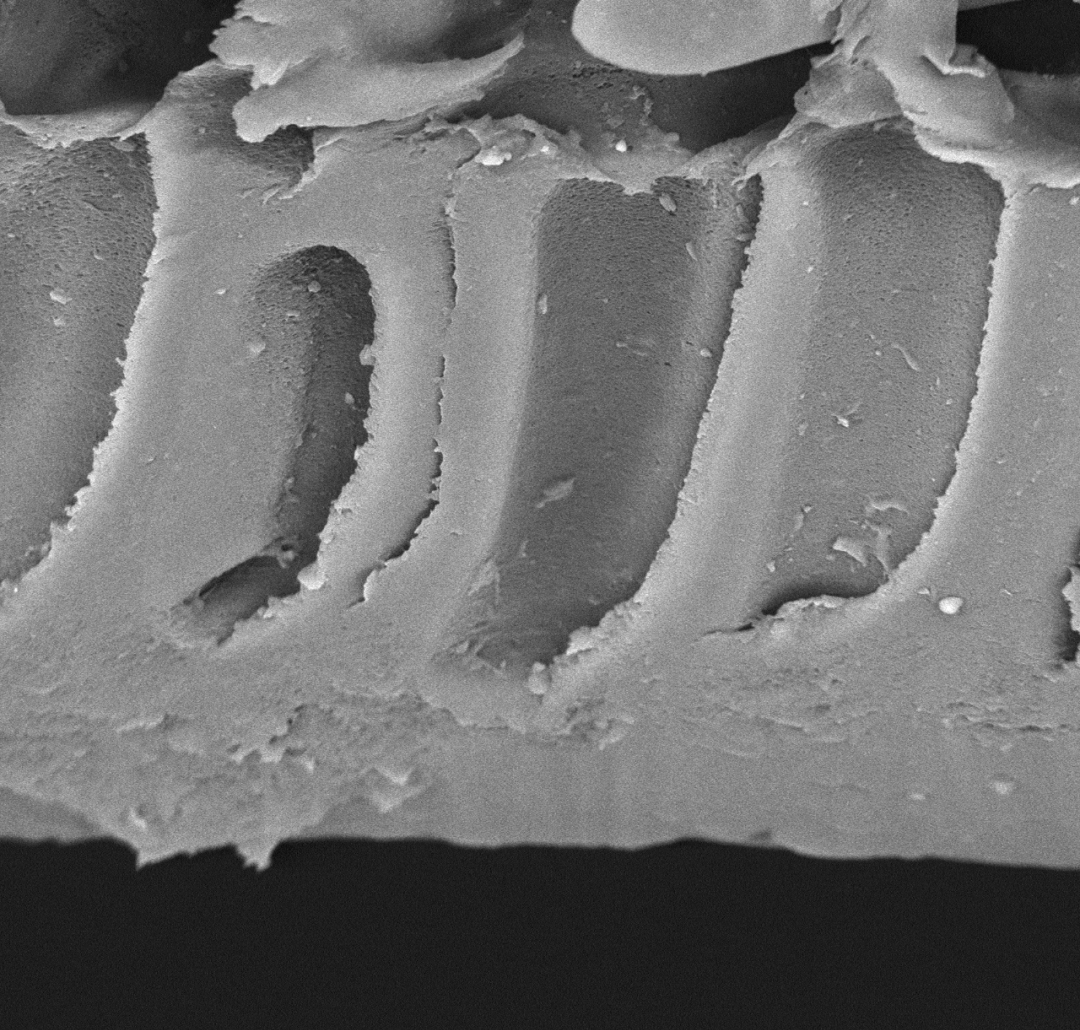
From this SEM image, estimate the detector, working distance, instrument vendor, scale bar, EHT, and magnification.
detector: BSE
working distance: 5.7 mm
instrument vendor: other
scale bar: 10000 nm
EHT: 15 kV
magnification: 3 K X